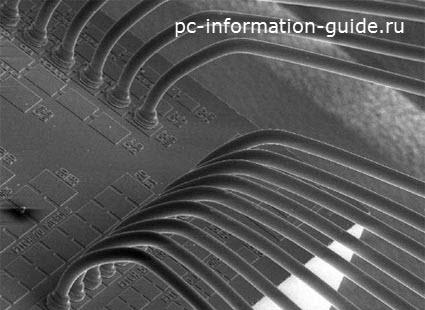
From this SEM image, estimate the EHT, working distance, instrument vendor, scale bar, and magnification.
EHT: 15 kV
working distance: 33 mm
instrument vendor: JEOL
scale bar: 200000 nm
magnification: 0.17 K X